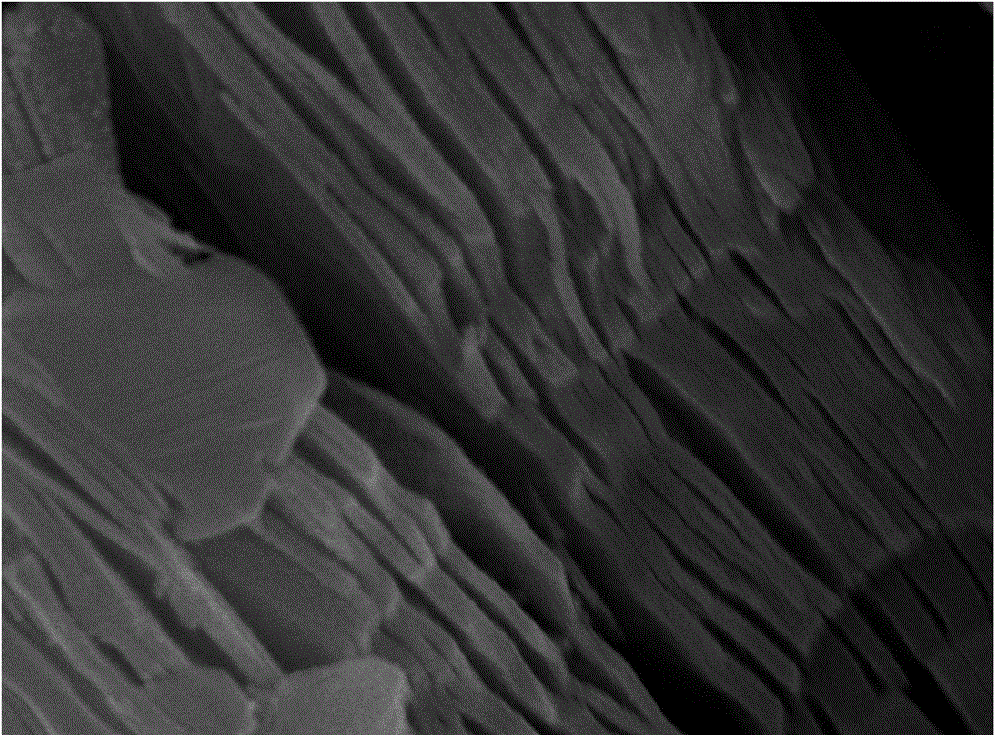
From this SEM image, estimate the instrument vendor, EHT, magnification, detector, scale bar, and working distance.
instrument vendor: JEOL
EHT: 15 kV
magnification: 50 K X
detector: SEI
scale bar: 100 nm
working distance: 7.6 mm